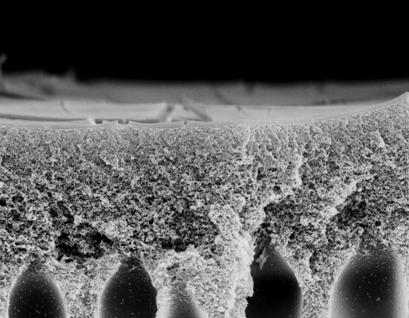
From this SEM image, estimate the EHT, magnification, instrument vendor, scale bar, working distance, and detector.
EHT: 20 kV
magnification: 0.5 K X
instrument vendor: Zeiss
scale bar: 2000 nm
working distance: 24 mm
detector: SE1